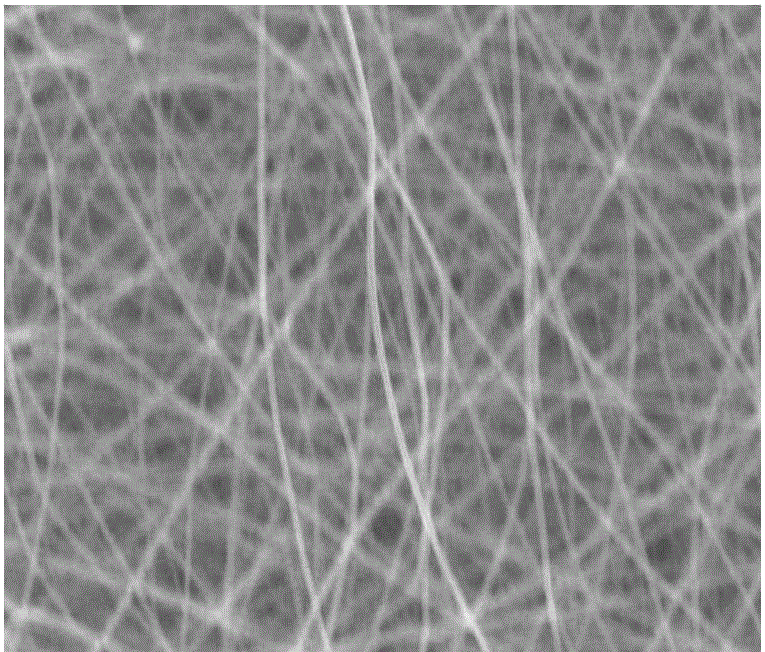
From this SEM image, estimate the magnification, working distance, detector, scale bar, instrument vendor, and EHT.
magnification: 1.5 K X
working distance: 11.5 mm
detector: ETD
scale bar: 40000 nm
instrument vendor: FEI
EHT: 30 kV